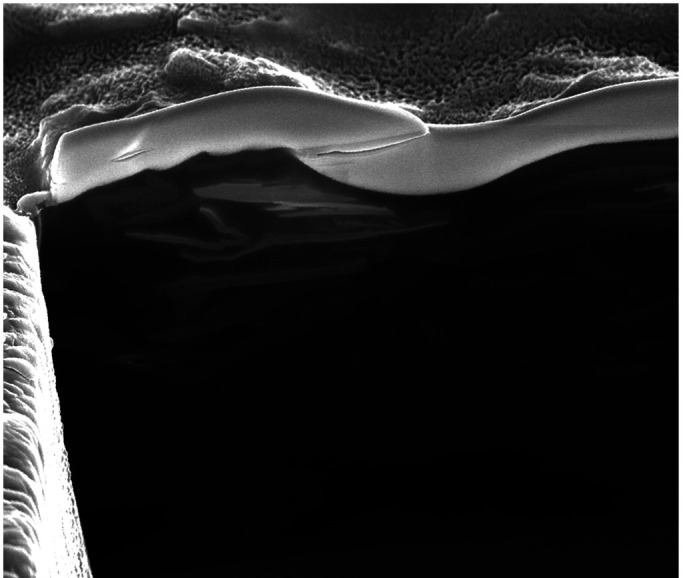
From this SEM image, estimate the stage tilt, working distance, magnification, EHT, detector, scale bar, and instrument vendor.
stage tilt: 52°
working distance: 5.1 mm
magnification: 20 K X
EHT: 15 kV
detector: SE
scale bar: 4000 nm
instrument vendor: FEI